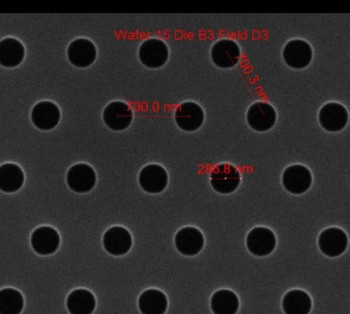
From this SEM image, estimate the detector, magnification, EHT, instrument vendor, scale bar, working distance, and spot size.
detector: ETD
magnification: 75 K X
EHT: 10 kV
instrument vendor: FEI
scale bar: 1000 nm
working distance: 10.2 mm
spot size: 1.5